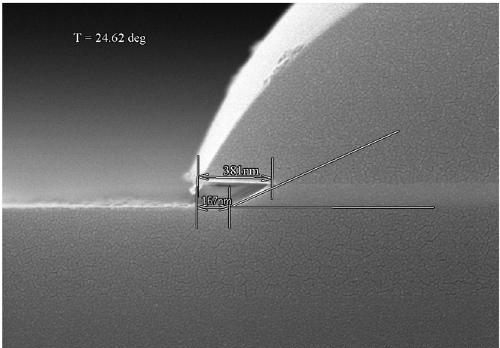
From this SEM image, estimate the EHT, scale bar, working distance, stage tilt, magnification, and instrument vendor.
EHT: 15 kV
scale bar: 1000 nm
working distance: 11.8 mm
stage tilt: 24.62°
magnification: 50 K X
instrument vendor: Hitachi